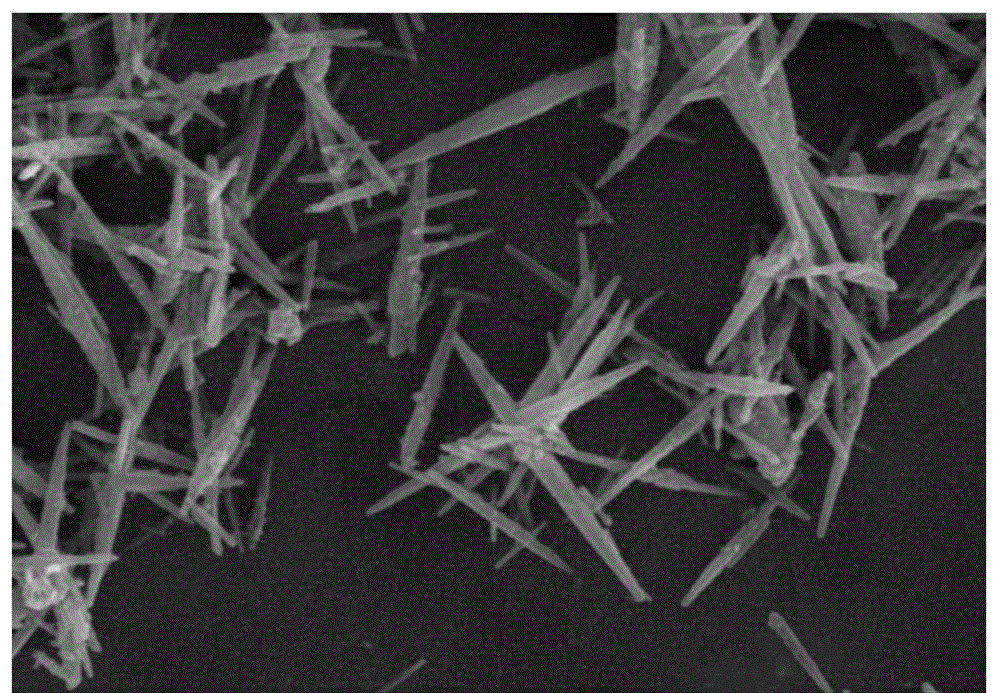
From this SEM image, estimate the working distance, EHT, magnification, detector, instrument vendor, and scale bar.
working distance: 6.7 mm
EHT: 15 kV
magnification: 10 K X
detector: SE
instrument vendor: Hitachi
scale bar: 5000 nm